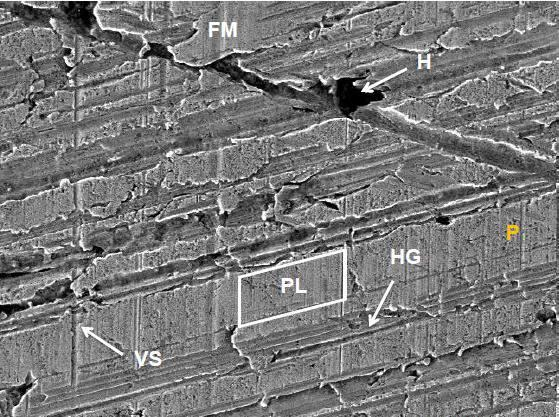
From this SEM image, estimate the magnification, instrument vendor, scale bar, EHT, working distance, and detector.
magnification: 1 K X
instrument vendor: FEI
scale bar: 50000 nm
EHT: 20 kV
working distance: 9.7 mm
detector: SE